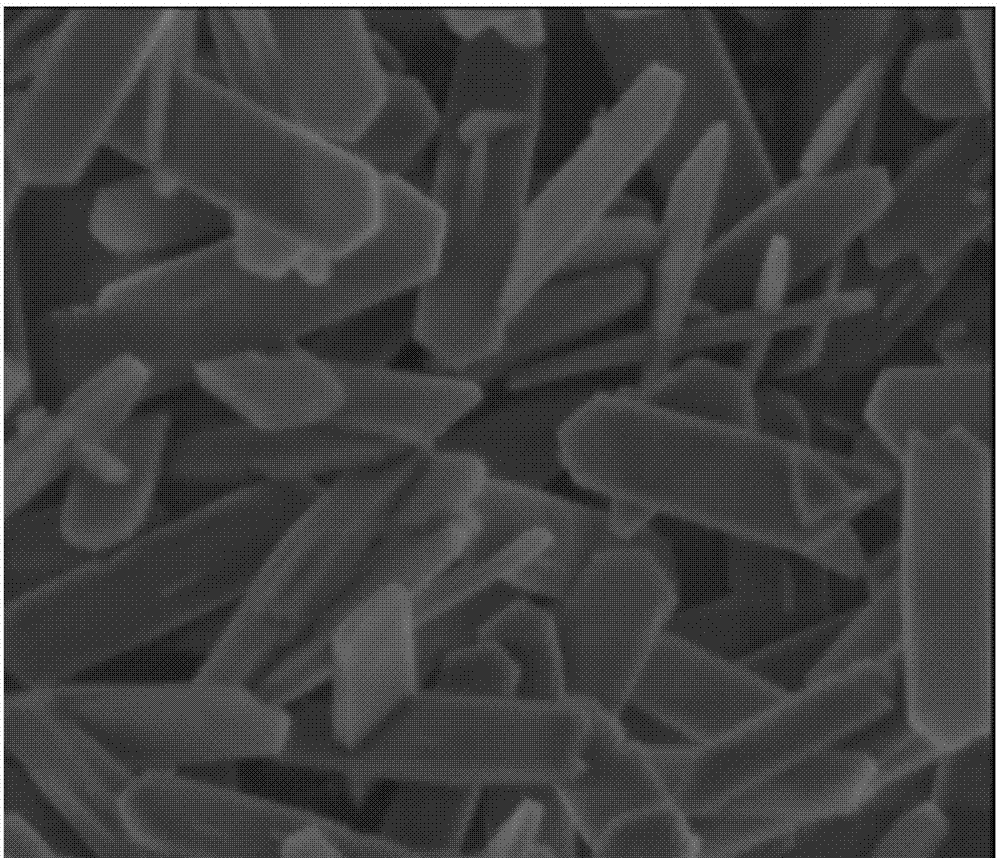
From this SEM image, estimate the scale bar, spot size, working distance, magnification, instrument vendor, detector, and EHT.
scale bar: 2000 nm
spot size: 3.5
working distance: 4.6 mm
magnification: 40 K X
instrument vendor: FEI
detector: ETD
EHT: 3 kV